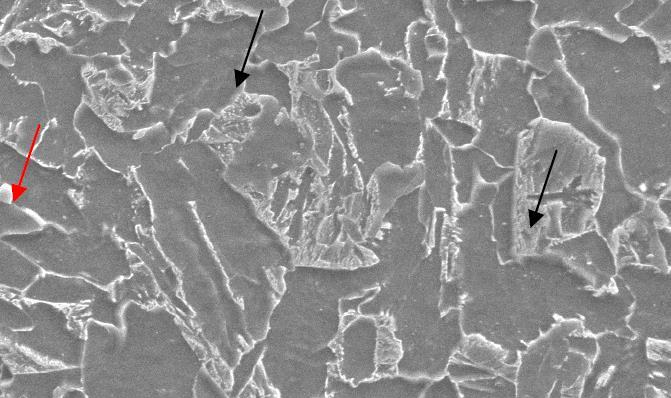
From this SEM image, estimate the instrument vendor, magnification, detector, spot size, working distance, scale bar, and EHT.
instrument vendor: FEI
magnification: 6.5 K X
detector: SE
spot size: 4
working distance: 10.1 mm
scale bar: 10000 nm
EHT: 20 kV